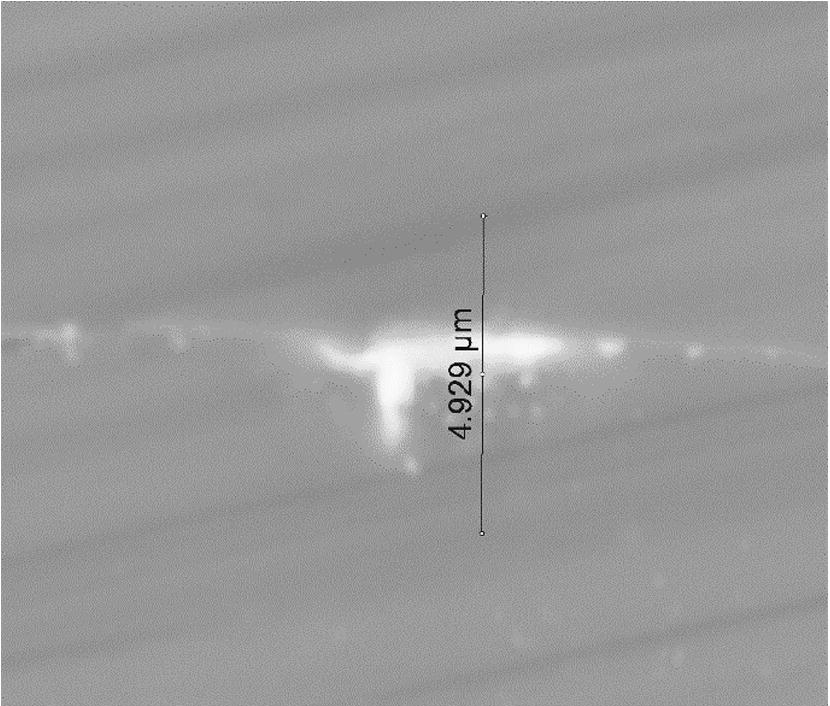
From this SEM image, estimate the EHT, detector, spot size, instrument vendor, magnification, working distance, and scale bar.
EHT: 20 kV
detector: BSED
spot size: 4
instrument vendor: FEI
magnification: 19.983 K X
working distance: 10 mm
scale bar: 4000 nm